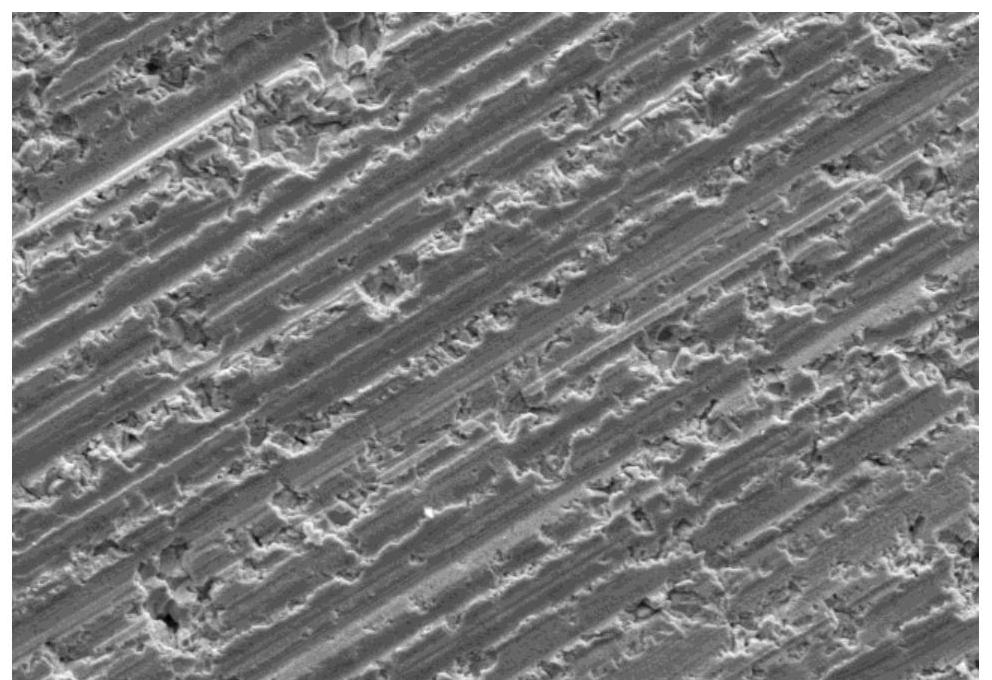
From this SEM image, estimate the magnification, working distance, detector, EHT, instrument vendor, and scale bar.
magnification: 0.5 K X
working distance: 8 mm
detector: LM(L)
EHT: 20 kV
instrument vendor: Hitachi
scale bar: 100000 nm